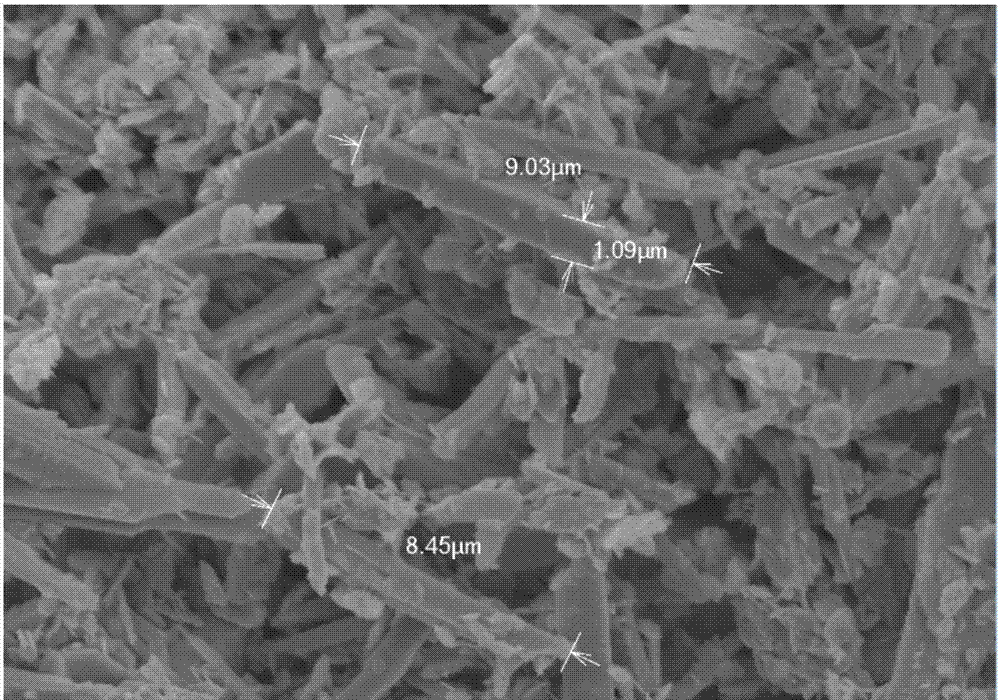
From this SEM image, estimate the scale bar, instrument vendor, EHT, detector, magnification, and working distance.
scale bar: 10000 nm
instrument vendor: Hitachi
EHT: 10 kV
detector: SE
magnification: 5 K X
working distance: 6.5 mm